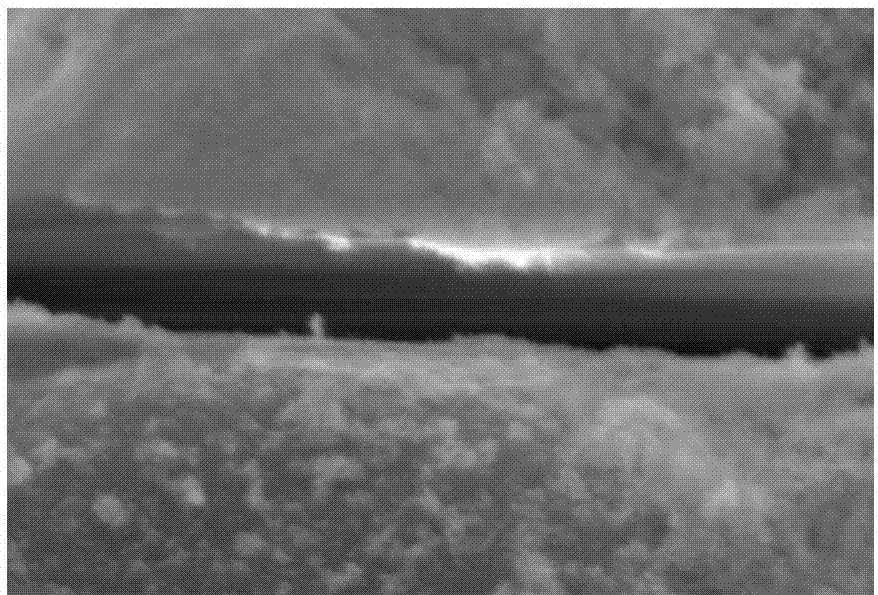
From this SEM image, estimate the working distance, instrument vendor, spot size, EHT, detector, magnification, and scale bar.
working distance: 24 mm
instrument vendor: JEOL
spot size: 33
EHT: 20 kV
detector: SEI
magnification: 10 K X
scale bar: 1000 nm